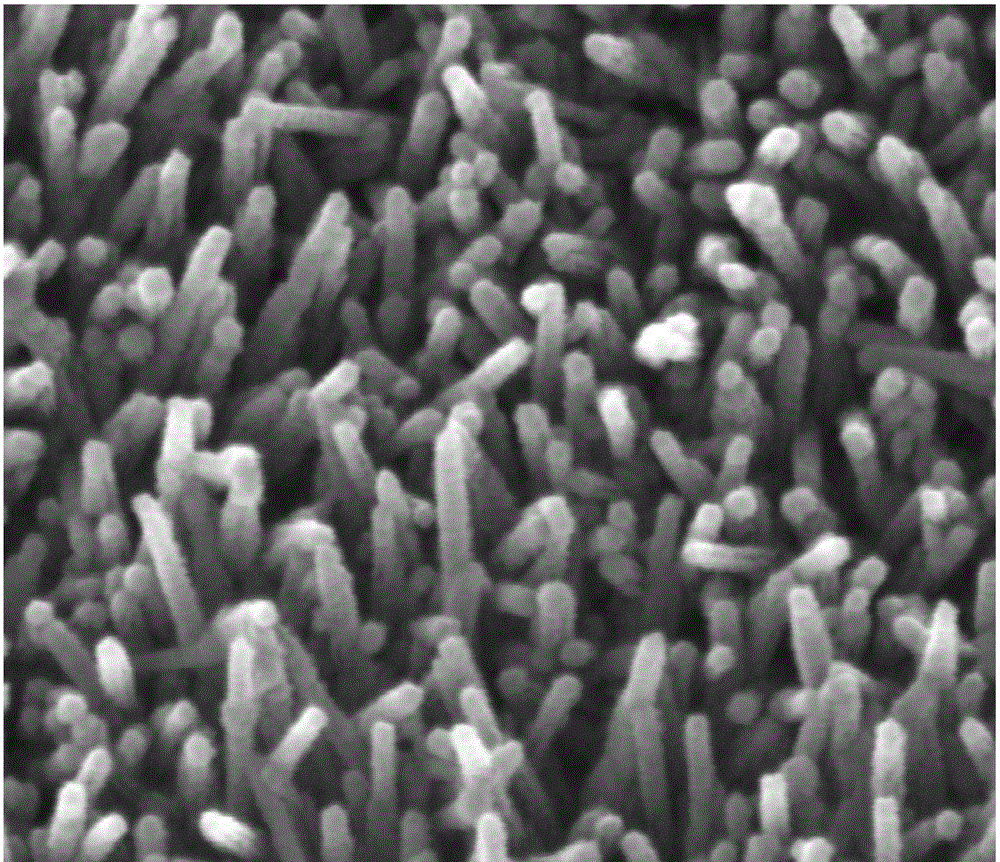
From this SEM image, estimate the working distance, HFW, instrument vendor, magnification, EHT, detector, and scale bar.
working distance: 9.4 mm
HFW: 1.28 µm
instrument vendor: FEI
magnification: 200 K X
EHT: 30 kV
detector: ETD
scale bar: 500 nm